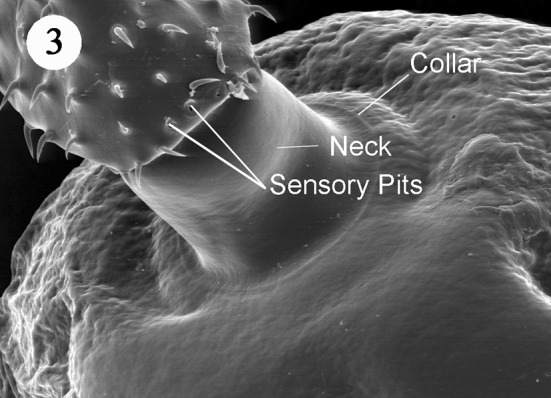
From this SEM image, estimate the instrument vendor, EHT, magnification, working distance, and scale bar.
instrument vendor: Zeiss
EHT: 15 kV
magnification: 0.3 K X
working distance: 10 mm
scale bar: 70000 nm